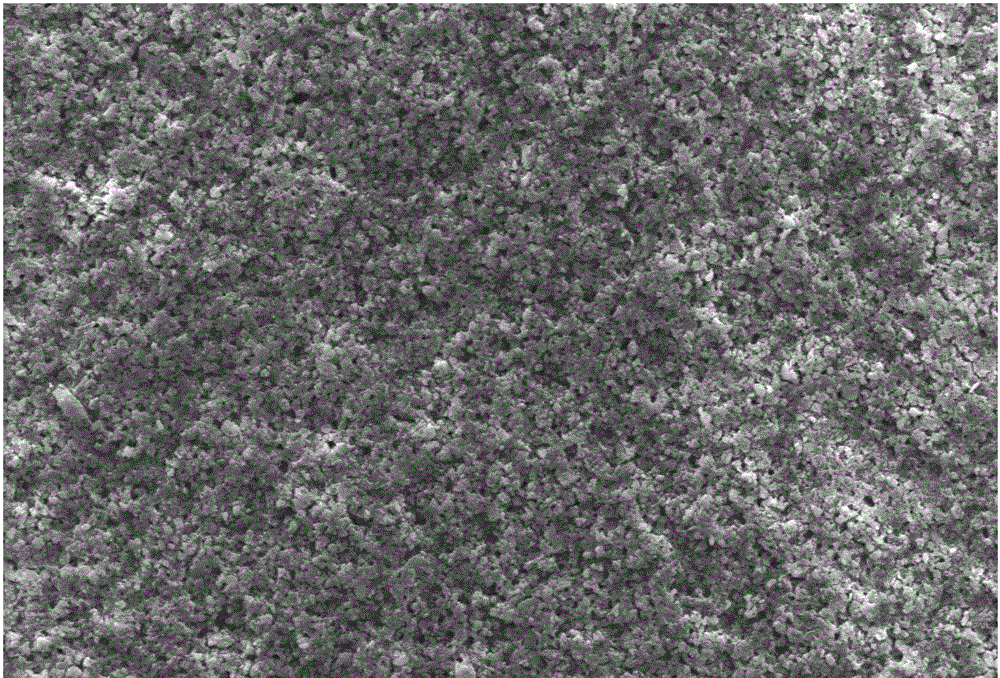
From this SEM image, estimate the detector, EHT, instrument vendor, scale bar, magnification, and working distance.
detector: SE1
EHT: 20 kV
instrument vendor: Zeiss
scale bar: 10000 nm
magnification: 0.5 K X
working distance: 8.5 mm